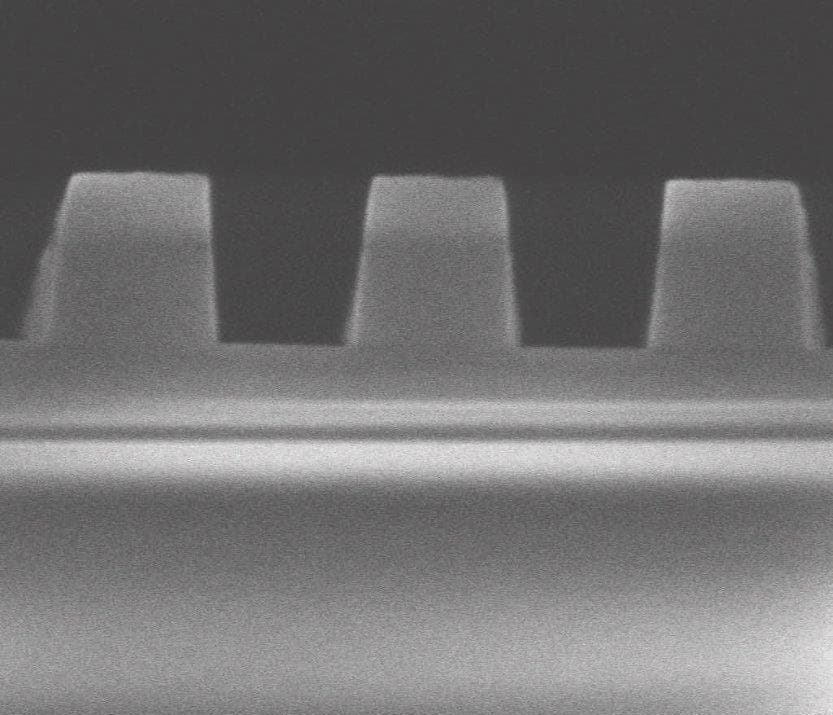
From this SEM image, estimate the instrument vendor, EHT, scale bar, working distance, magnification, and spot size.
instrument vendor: FEI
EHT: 5 kV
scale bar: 500 nm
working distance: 3.8 mm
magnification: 250 K X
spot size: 3